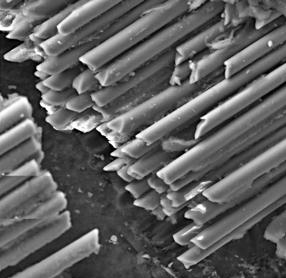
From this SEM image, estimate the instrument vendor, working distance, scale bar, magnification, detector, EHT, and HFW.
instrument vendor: Tescan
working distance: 11.61 mm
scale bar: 50000 nm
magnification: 1.55 K X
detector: SE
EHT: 24 kV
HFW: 223 µm